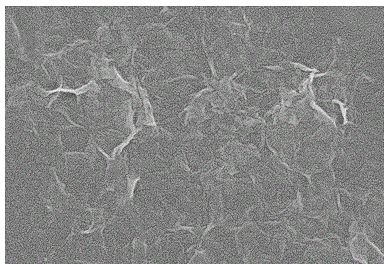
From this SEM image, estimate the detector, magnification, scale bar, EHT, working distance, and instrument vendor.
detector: SE(M)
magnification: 30 K X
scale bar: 1000 nm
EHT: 7 kV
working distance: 8.9 mm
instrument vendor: Hitachi